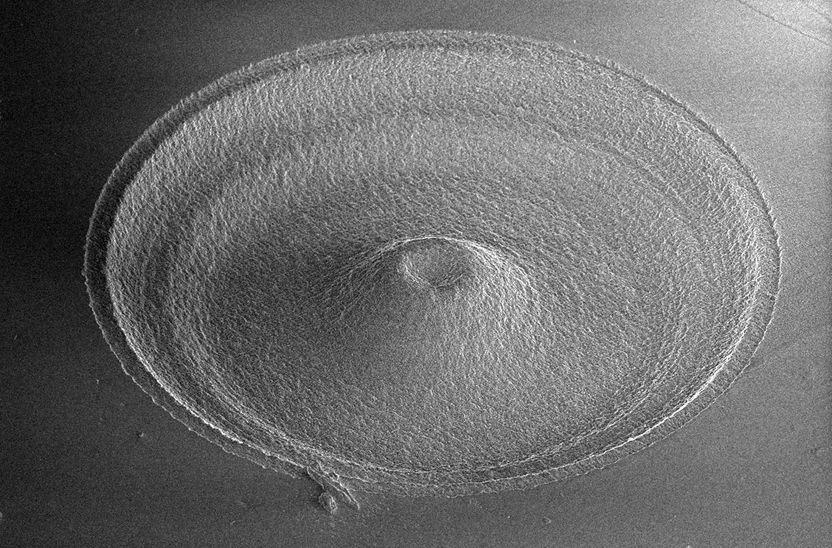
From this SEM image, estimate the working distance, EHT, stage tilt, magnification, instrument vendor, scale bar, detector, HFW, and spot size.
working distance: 4.9 mm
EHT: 3 kV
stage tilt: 49°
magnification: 0.3 K X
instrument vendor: FEI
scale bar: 100000 nm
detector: ETD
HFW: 423 µm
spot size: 2.5